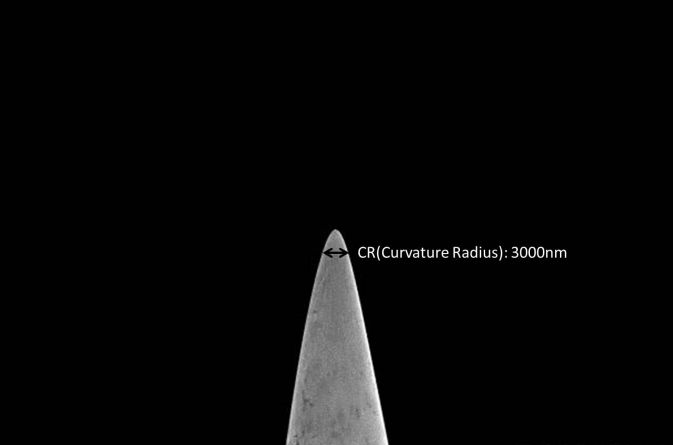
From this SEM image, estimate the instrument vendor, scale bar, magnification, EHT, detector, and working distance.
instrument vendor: Hitachi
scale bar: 50000 nm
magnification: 0.6 K X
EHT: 2 kV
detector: SE(M)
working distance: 6 mm